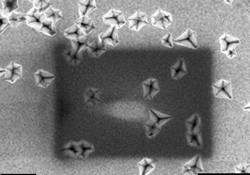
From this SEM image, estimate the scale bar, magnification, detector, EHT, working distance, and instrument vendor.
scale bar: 100 nm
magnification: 50 K X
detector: InLens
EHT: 2 kV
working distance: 4 mm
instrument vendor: Zeiss